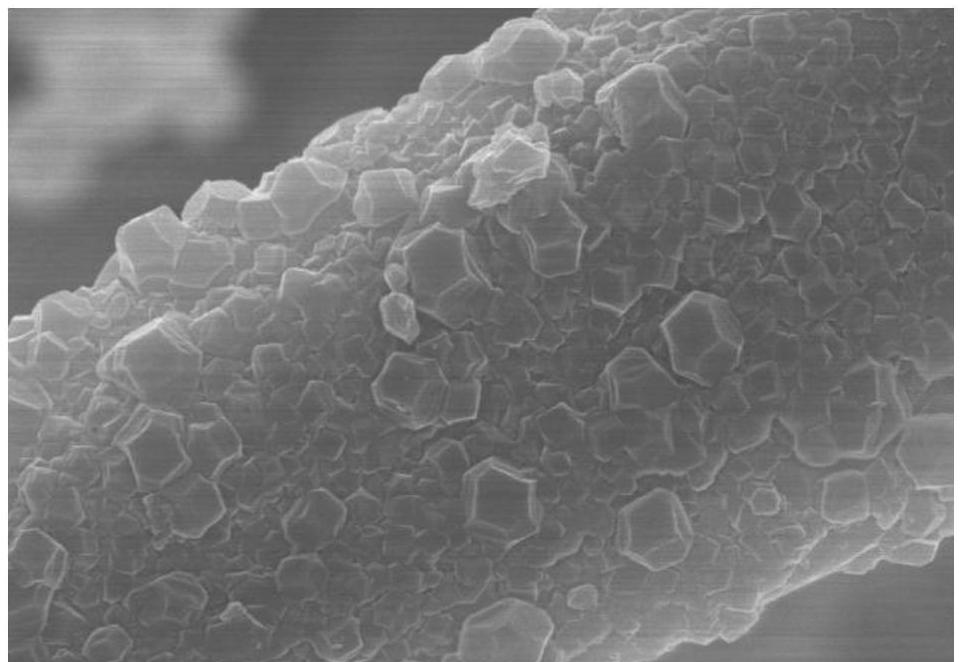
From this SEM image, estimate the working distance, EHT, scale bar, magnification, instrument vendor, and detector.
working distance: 13.4 mm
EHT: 10 kV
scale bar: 2000 nm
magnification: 20 K X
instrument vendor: Hitachi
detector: SE(U)